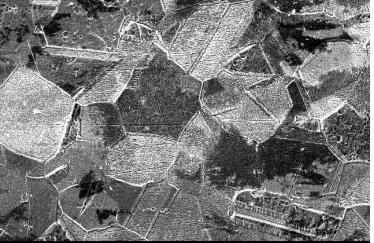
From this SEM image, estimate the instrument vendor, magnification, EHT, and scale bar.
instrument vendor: JEOL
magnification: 0.5 K X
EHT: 20 kV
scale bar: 50000 nm